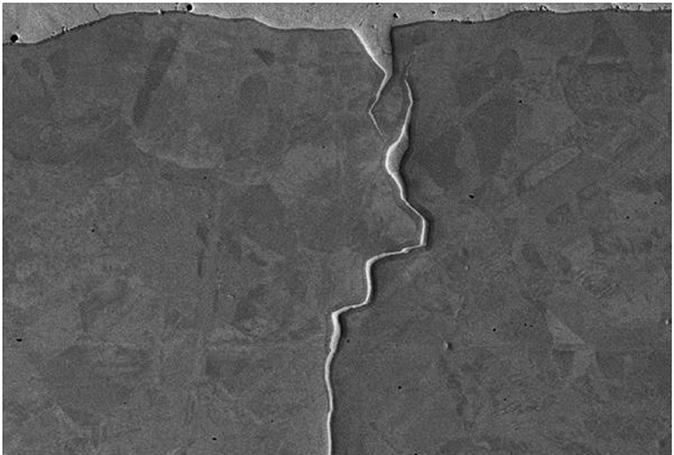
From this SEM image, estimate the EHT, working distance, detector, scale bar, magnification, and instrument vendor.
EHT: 20 kV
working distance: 13 mm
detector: SE2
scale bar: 10000 nm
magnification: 1 K X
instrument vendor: Zeiss